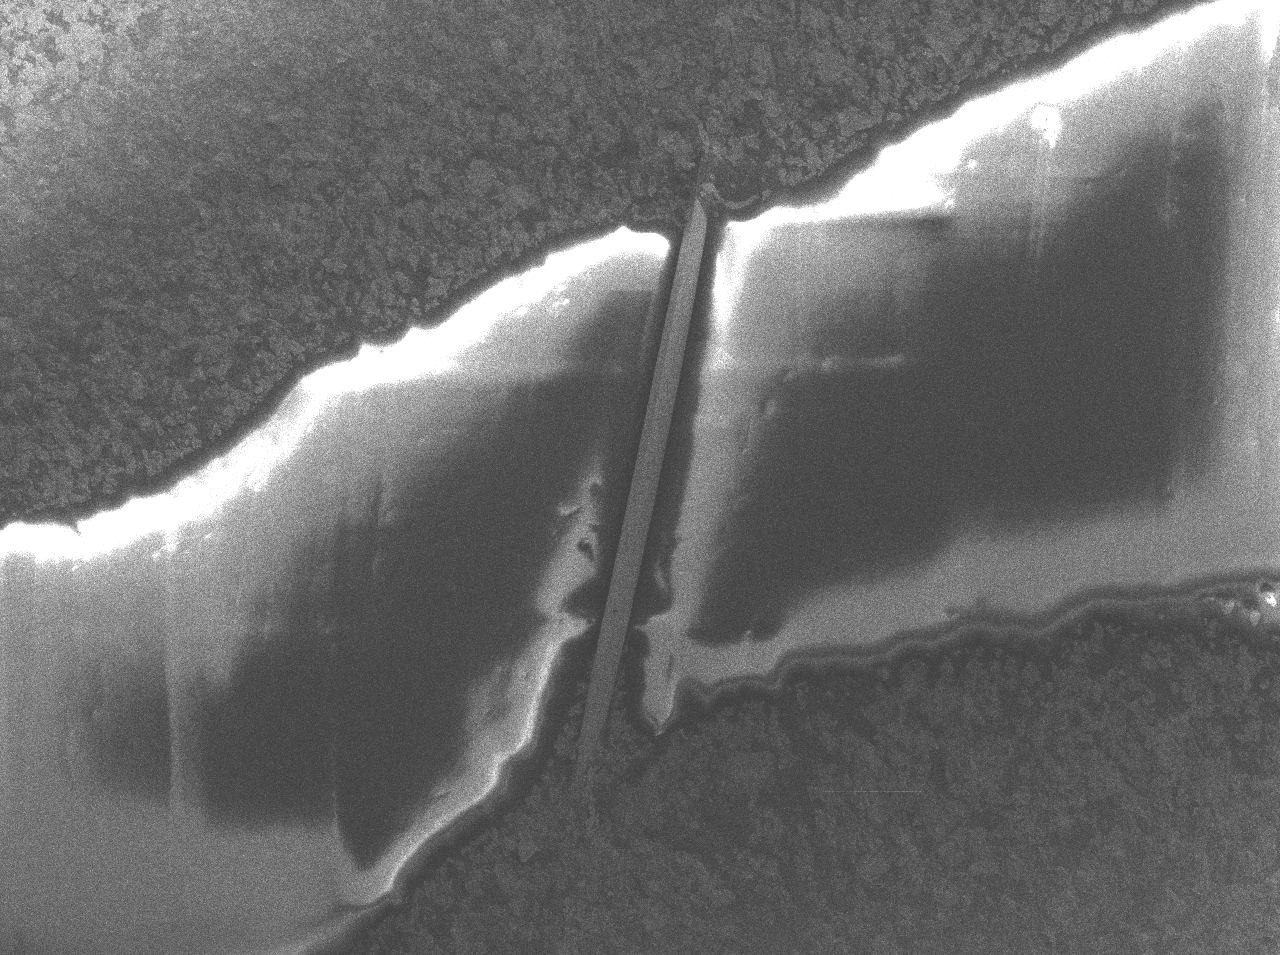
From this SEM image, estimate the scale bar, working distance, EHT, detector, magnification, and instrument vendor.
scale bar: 200 nm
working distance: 11.8 mm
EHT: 2 kV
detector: SEI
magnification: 2.7 K X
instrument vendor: JEOL